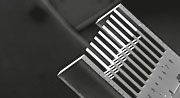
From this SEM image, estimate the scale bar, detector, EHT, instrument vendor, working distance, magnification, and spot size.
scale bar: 500000 nm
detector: SE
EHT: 15 kV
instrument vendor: FEI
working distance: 17.2 mm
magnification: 0.046 K X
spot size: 3